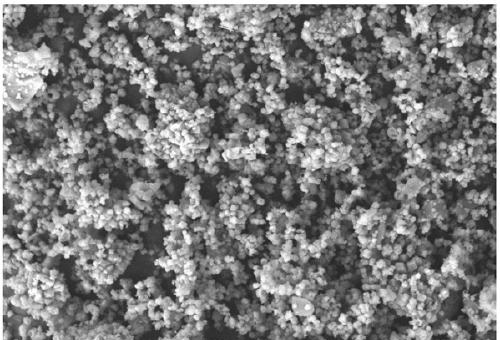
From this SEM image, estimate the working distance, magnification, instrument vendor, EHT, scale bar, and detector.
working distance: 7.8 mm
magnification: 5 K X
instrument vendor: Zeiss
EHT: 10 kV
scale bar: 2000 nm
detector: SE2